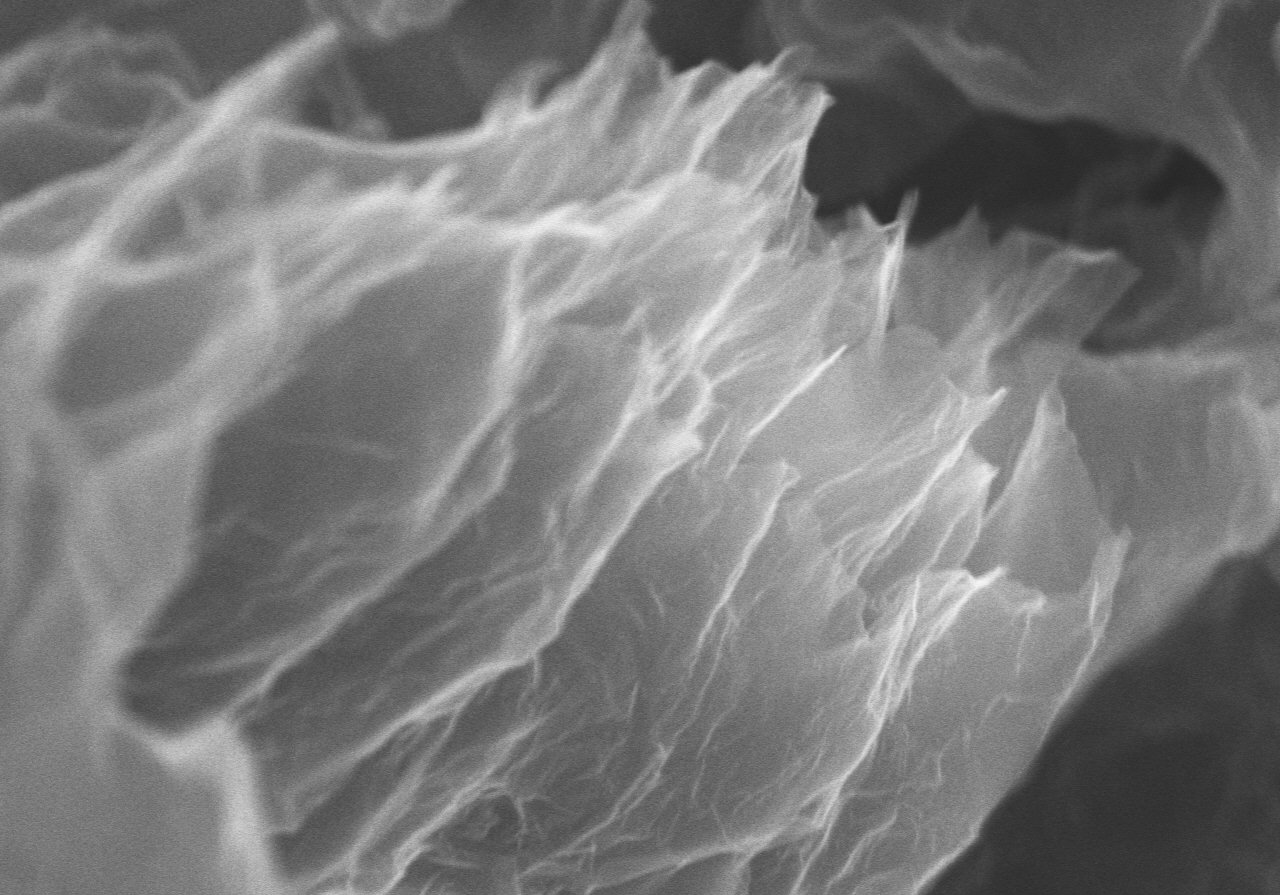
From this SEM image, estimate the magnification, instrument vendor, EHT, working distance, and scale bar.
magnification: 25 K X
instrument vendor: Hitachi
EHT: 10 kV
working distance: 8.4 mm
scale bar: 2000 nm